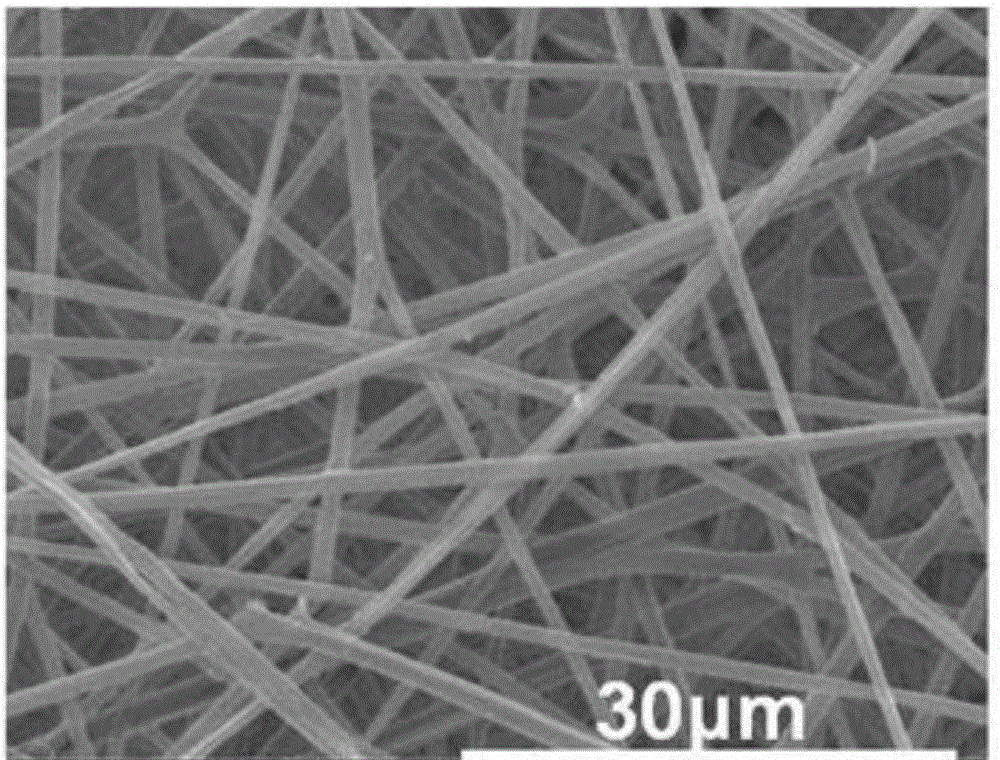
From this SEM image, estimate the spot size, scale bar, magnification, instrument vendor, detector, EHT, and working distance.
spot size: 4.5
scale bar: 30000 nm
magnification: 5 K X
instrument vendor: FEI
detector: ETD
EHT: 10 kV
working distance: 10.6 mm